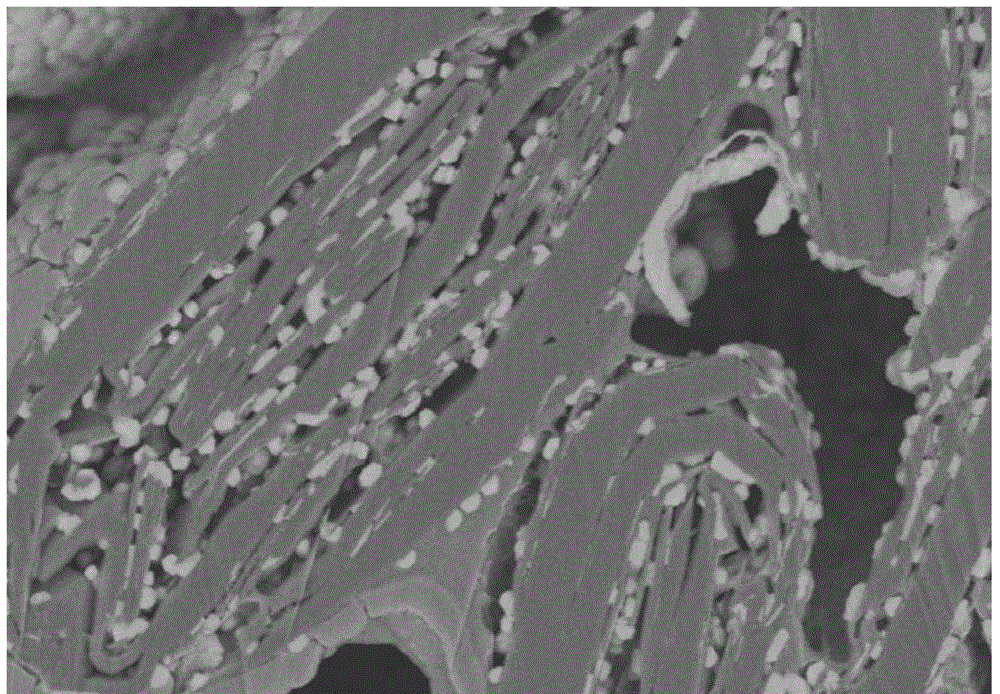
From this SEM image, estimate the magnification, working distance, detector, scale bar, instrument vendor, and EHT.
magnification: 10 K X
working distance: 5 mm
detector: SE(U,LA100)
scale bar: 5000 nm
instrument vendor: Hitachi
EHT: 3 kV